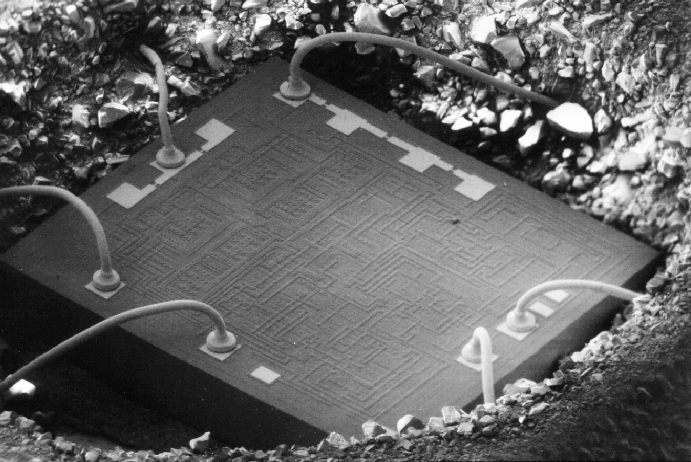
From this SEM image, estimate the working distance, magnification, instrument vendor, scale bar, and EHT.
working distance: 25 mm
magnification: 0.05 K X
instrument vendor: JEOL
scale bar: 100000 nm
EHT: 5 kV